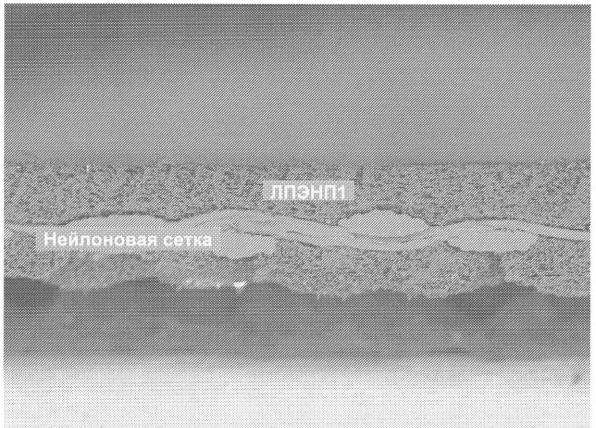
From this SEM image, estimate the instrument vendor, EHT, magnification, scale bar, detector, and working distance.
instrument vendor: JEOL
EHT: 10 kV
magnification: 0.1 K X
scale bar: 100000 nm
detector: COMPO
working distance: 14 mm